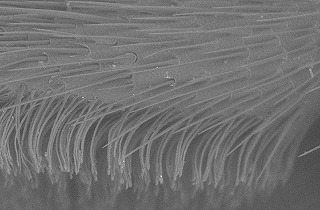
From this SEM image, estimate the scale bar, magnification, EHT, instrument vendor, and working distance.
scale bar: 200000 nm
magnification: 0.25 K X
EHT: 15 kV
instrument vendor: Hitachi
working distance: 13.3 mm